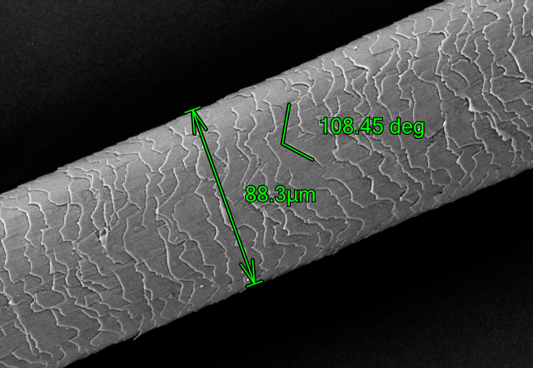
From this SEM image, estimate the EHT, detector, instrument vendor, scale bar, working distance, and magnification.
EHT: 10 kV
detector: BSE L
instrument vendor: Hitachi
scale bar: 100000 nm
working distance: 5.7 mm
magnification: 0.5 K X